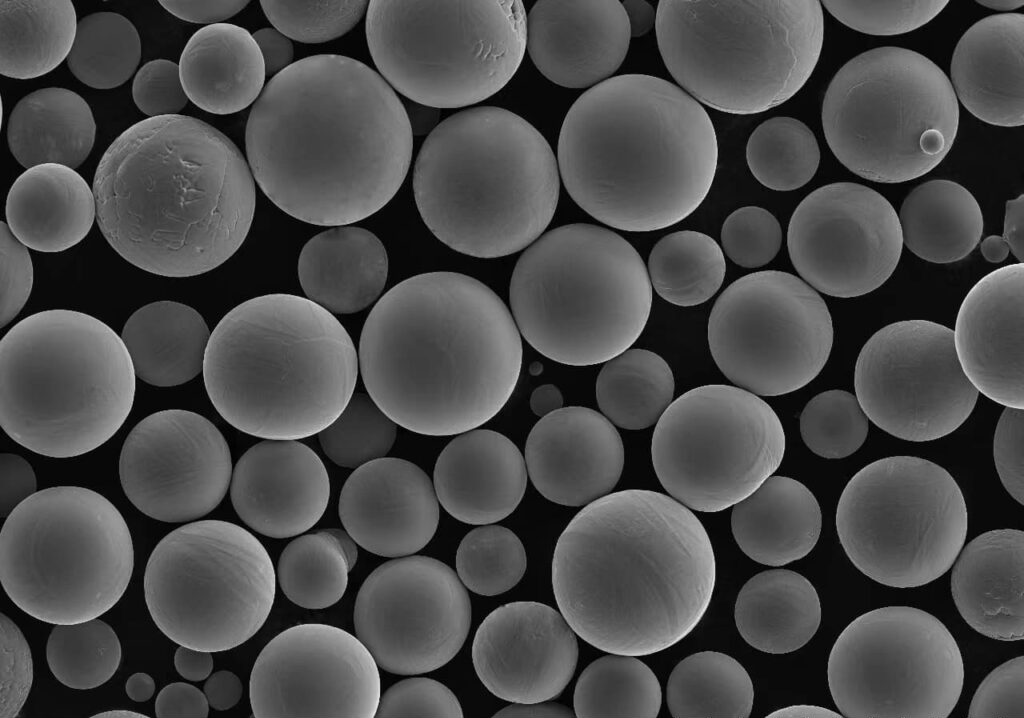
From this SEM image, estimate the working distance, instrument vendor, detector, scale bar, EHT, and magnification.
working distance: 7.6 mm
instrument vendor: Hitachi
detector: SE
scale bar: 200000 nm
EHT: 15 kV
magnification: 0.2 K X